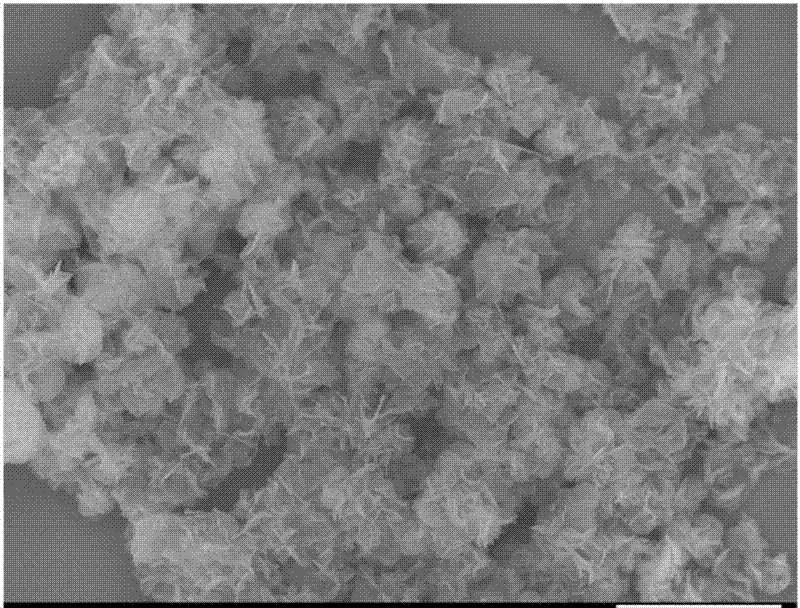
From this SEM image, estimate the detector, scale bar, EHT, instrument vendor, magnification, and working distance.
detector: SEI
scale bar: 10000 nm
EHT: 10 kV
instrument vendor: JEOL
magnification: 2.5 K X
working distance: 7.1 mm